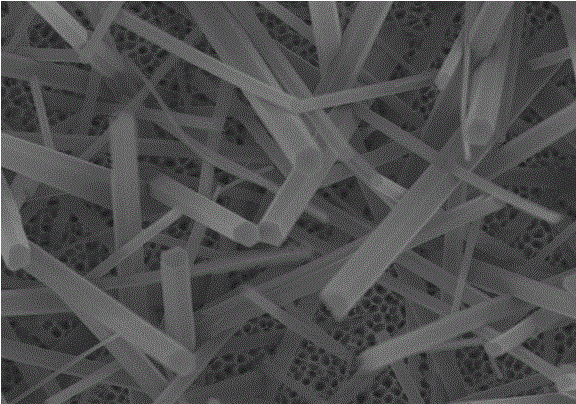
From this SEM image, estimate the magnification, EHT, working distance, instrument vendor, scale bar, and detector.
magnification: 10 K X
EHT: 5 kV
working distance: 6.4 mm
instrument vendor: Hitachi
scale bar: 5000 nm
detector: SE(M)